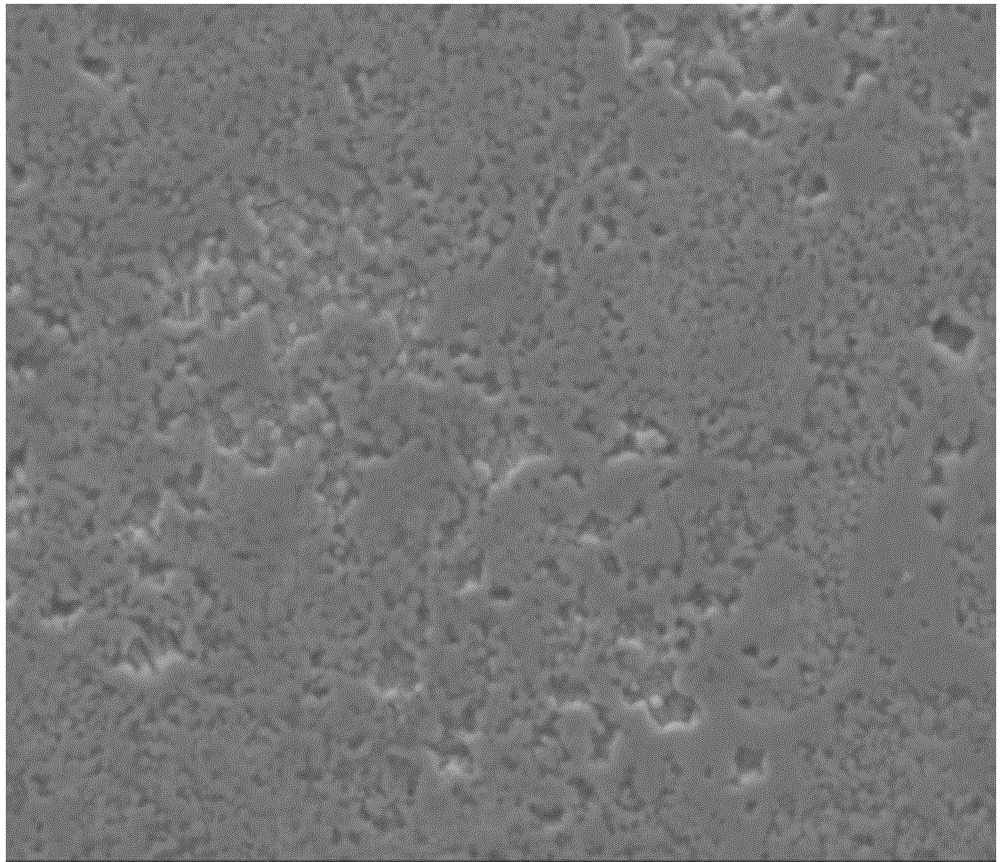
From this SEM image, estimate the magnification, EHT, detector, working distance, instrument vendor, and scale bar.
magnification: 5 K X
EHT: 15 kV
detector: ETD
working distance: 13.4 mm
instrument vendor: FEI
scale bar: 20000 nm